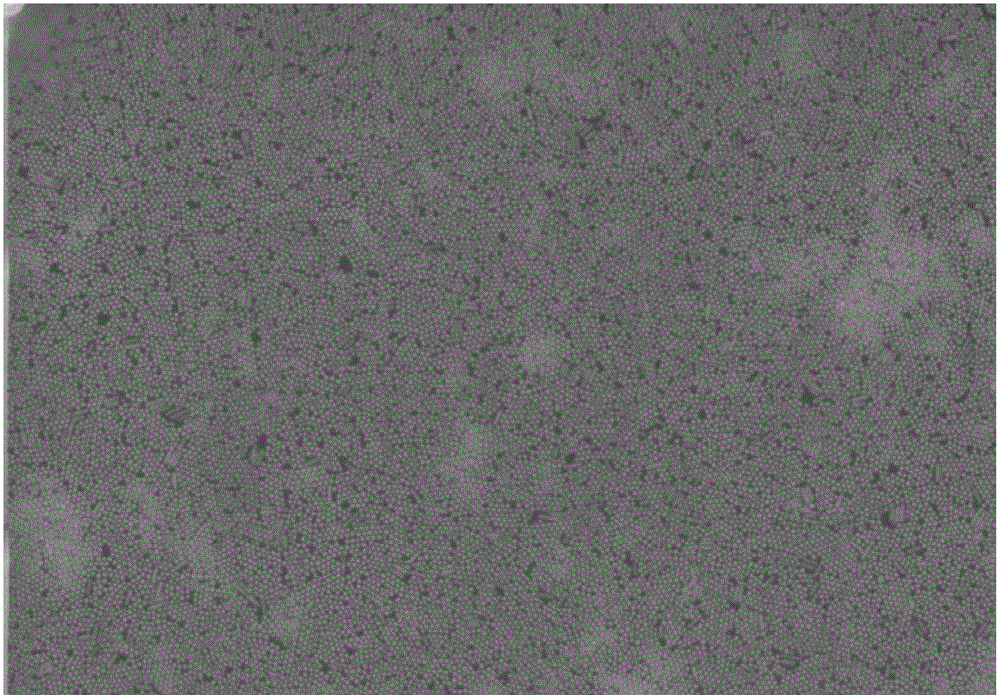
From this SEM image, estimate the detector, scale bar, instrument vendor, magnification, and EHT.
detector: SE(M)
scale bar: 1000 nm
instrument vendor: Hitachi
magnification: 35 K X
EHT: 10 kV